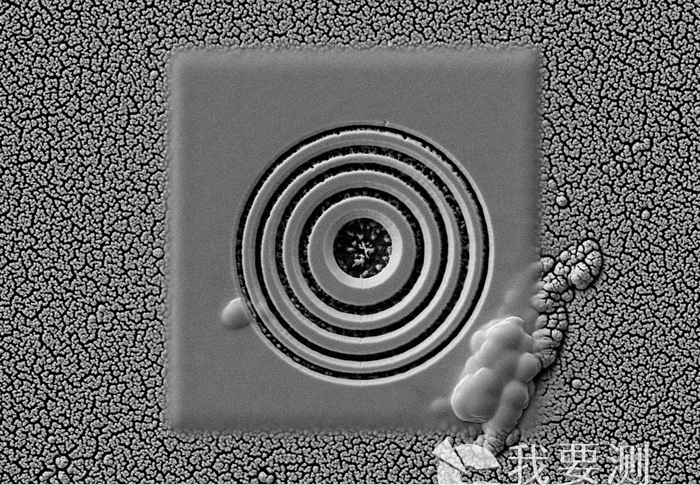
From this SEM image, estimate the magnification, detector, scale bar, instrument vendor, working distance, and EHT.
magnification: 5 K X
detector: SE2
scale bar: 1000 nm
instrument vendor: Zeiss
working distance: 8.3 mm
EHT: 5 kV